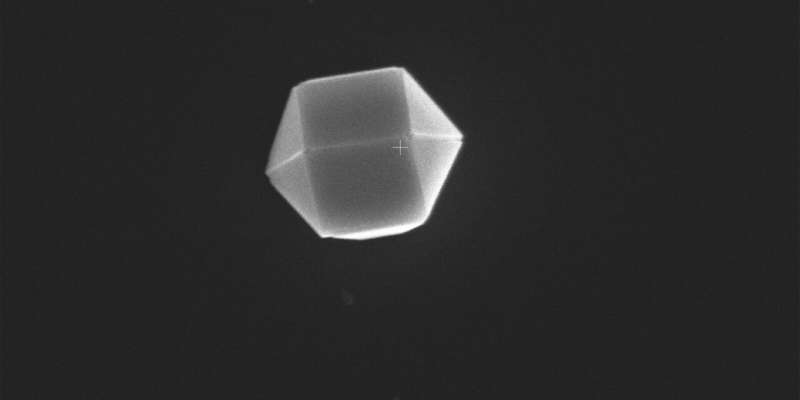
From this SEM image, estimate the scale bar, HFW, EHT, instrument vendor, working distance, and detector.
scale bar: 1000 nm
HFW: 4.14 µm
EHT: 5 kV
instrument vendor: Thermo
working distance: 9.9 mm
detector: T2